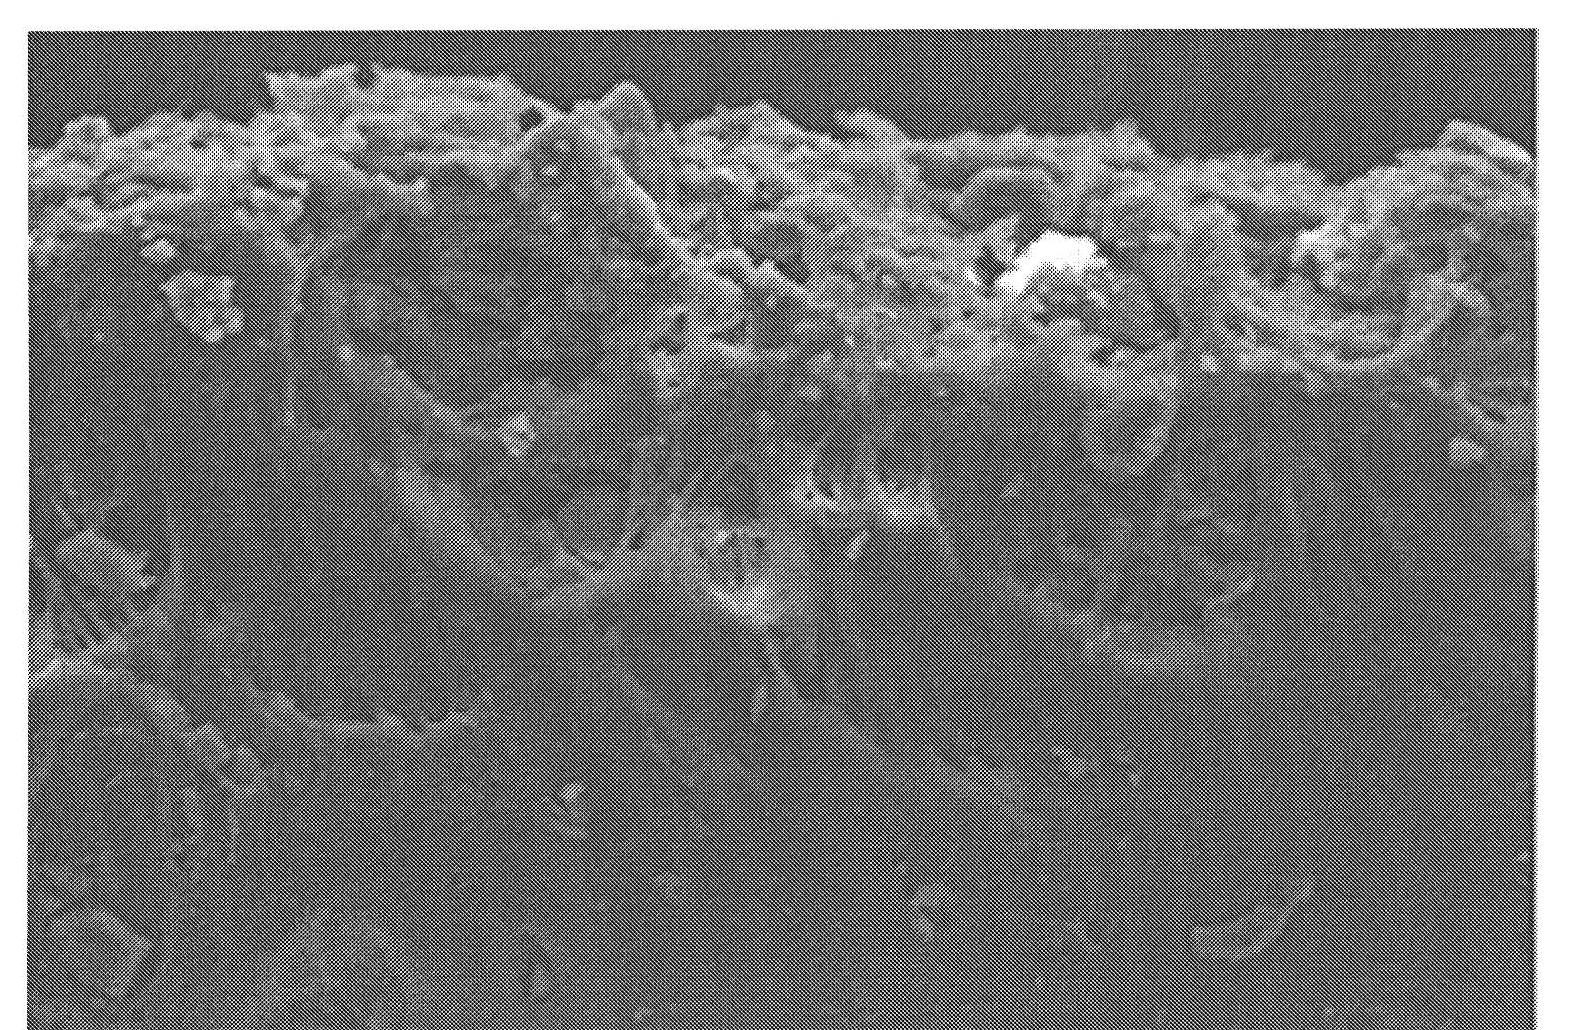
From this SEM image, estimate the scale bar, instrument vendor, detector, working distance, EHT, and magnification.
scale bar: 100000 nm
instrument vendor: Hitachi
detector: SE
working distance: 15 mm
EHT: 20 kV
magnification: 0.3 K X